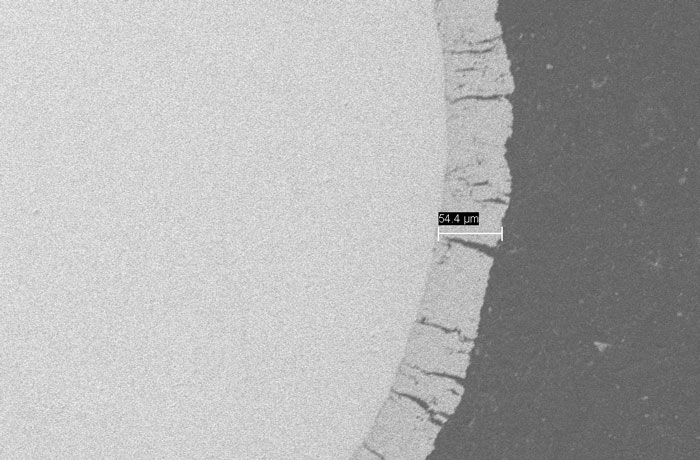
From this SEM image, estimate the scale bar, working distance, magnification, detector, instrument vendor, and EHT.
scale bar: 20000 nm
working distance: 12 mm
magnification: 0.5 K X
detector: SE1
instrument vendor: Zeiss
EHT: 15 kV